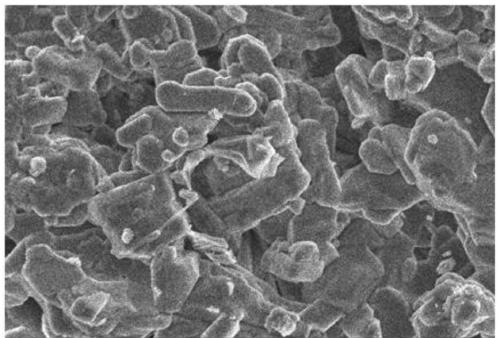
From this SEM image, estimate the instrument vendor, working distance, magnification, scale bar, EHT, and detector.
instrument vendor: JEOL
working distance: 11 mm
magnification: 2 K X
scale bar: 10000 nm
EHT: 10 kV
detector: SEI